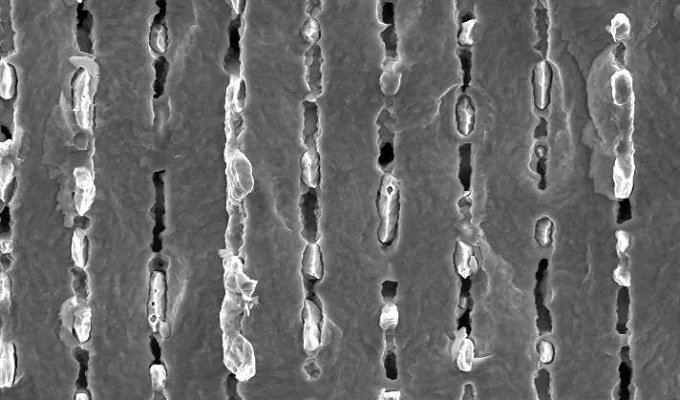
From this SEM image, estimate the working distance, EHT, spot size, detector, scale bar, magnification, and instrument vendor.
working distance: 9 mm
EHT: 10 kV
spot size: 3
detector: SE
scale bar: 10000 nm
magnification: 2.5 K X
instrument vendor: FEI